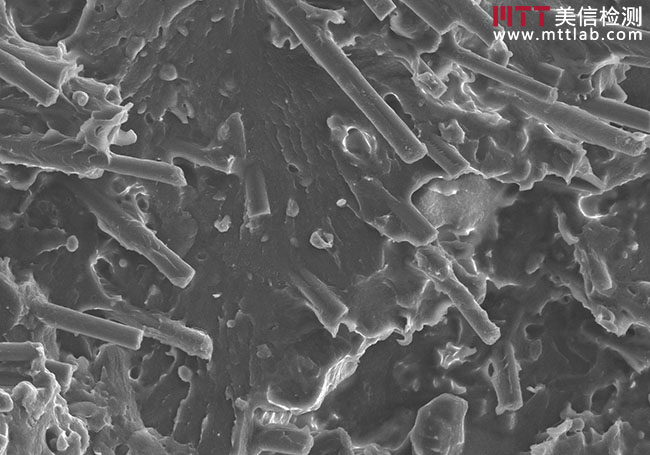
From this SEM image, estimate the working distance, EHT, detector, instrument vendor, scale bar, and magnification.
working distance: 44.1 mm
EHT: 15 kV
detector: SE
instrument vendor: Hitachi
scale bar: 100000 nm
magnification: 0.5 K X